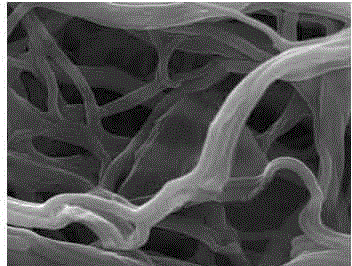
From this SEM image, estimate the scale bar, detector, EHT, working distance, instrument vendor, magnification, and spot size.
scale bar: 10000 nm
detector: ETD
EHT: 30 kV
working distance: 10.1 mm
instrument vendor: FEI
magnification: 10 K X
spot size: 3